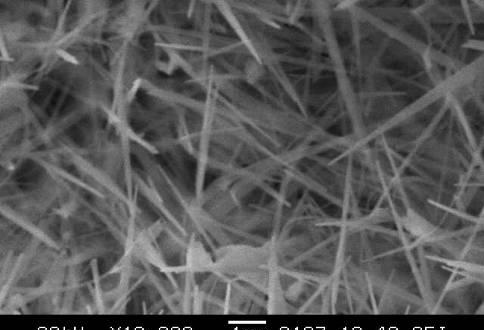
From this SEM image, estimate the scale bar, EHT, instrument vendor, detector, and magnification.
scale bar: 1000 nm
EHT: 20 kV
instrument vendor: JEOL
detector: SEI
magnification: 10 K X